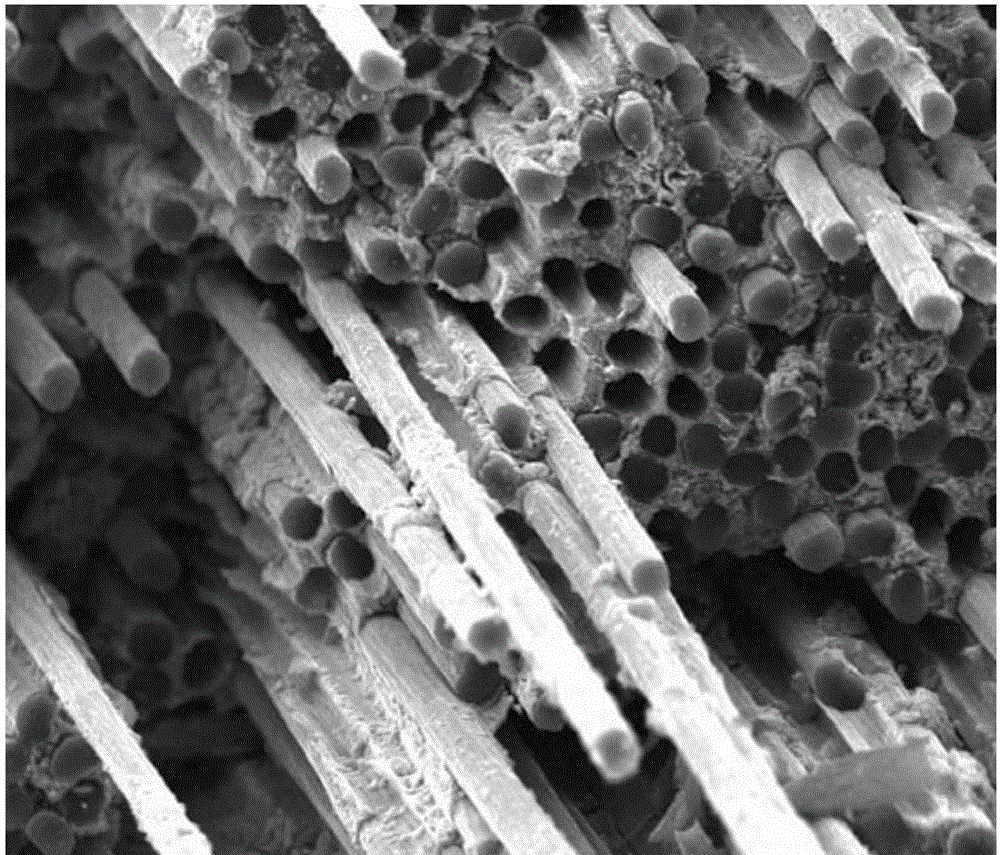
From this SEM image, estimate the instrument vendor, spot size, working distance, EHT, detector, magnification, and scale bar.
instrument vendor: FEI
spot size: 3.5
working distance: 14.5 mm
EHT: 20 kV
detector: ETD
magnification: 1 K X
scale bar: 40000 nm